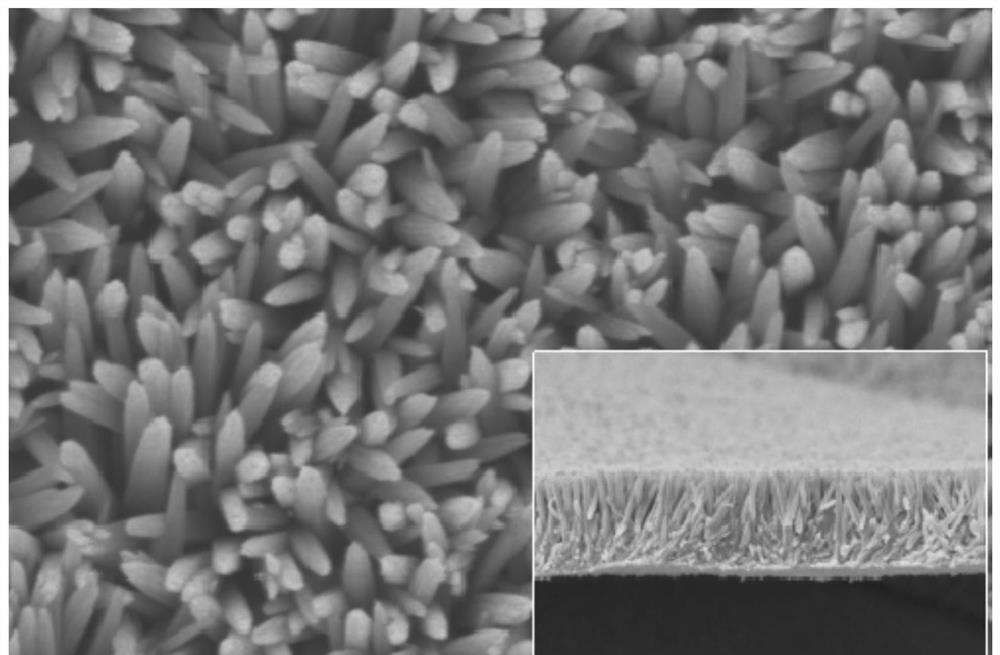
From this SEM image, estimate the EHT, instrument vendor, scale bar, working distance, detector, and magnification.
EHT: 3 kV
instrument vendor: Hitachi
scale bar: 1000 nm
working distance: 8.5 mm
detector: SE(U)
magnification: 50 K X